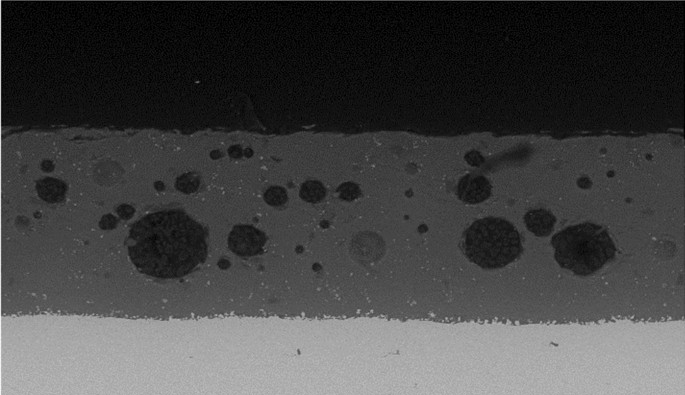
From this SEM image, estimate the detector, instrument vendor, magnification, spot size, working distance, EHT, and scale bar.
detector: BSE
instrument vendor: FEI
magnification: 0.3 K X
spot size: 3.7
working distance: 9.8 mm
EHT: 20 kV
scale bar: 100000 nm